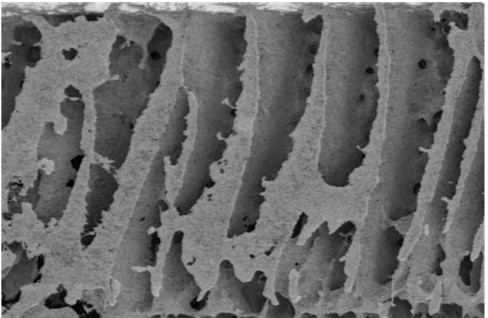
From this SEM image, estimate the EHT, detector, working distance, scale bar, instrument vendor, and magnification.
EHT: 1 kV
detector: SE(M)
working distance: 7.7 mm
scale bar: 20000 nm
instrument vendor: Hitachi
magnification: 2 K X